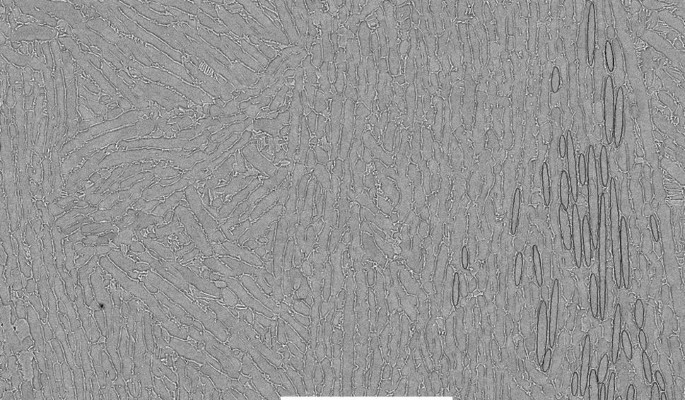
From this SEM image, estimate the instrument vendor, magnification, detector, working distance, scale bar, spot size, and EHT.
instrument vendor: FEI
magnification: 1 K X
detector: BSE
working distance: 10 mm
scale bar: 50000 nm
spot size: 3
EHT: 15 kV